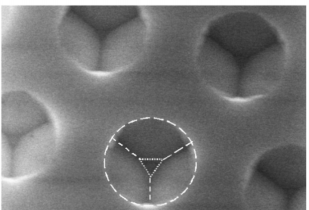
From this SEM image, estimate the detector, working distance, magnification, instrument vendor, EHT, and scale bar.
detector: SE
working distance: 15.6 mm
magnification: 10 K X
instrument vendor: Hitachi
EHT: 15 kV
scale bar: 5000 nm